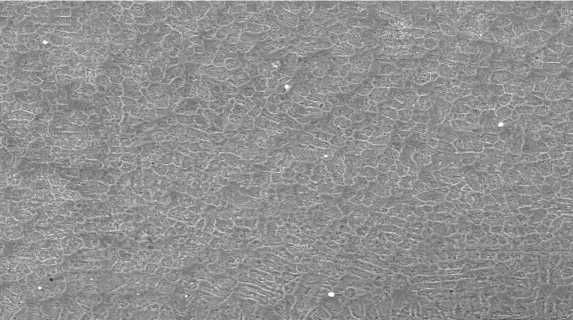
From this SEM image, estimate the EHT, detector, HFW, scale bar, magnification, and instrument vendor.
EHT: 20 kV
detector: SE Detector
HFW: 150 µm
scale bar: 20000 nm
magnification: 2 K X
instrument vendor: Tescan